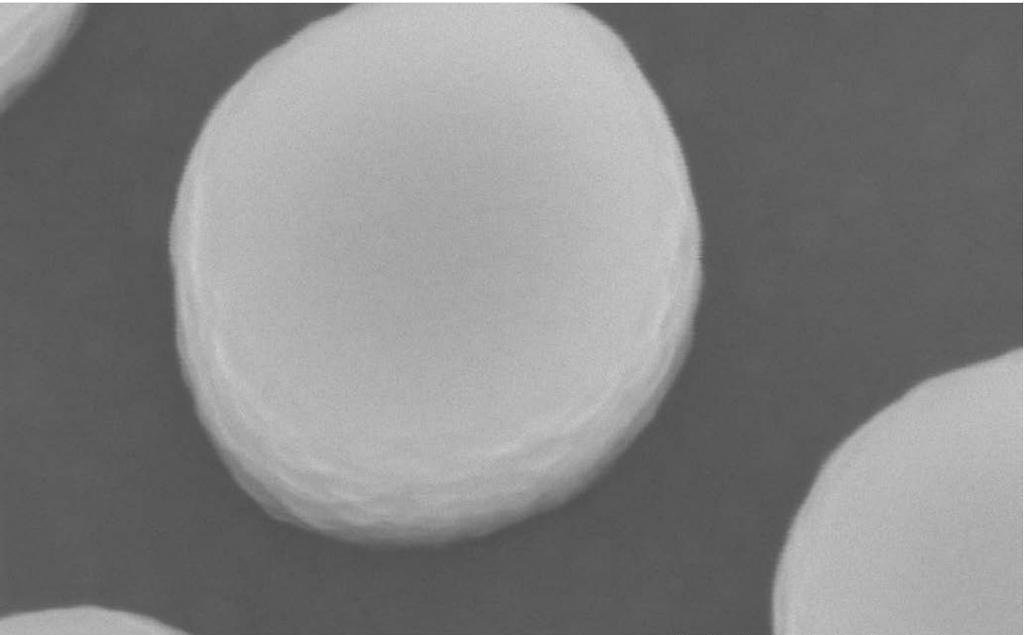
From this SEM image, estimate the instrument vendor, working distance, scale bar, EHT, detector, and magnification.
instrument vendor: Hitachi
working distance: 3.3 mm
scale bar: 200 nm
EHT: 5 kV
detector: SE(U)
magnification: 200 K X